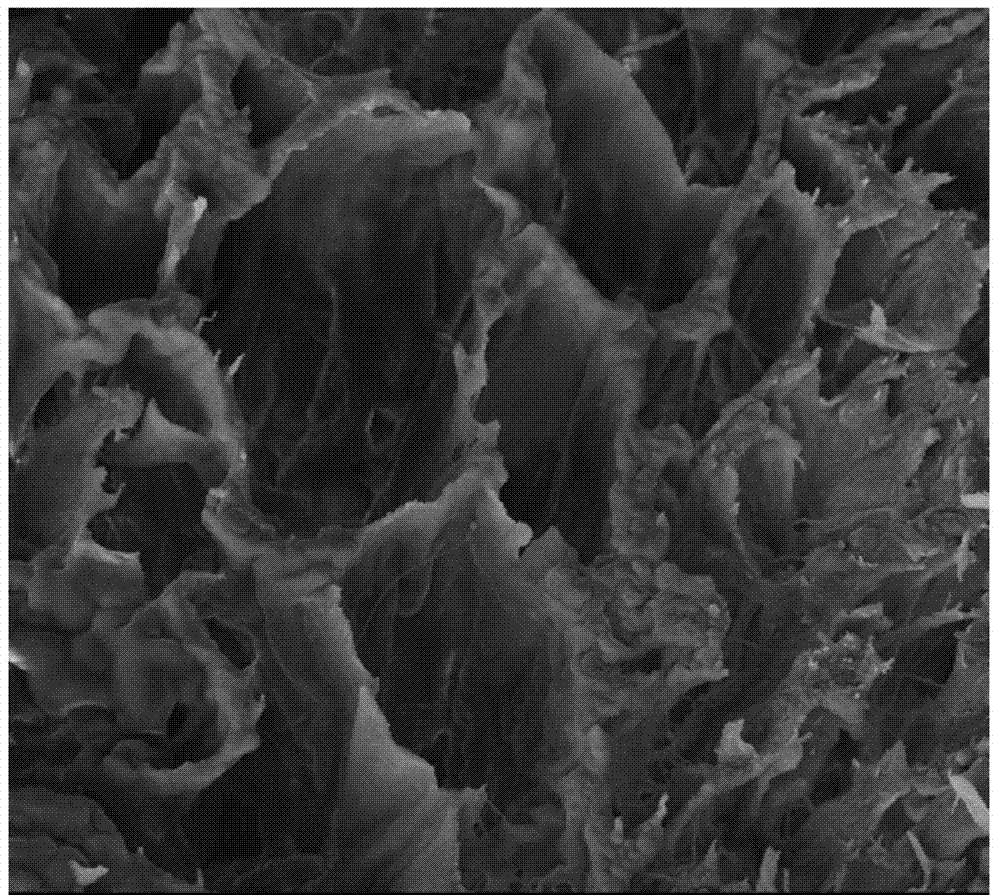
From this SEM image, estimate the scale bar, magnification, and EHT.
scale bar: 100000 nm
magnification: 0.4 K X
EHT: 20 kV